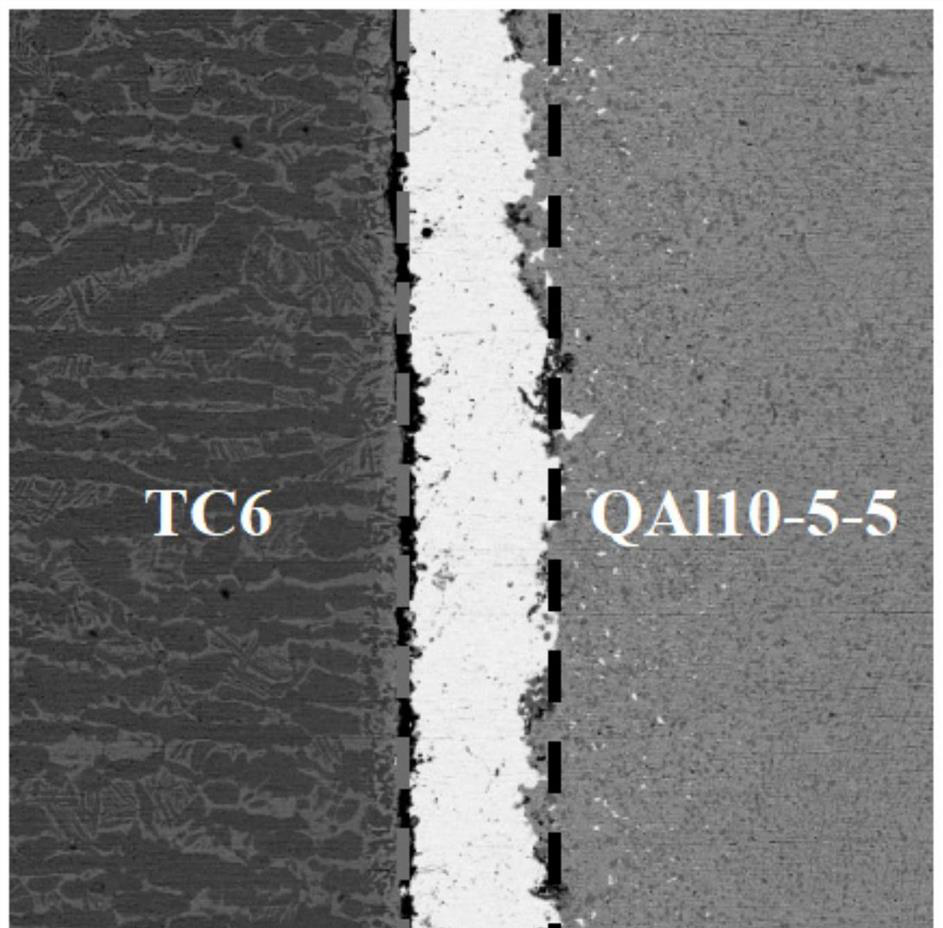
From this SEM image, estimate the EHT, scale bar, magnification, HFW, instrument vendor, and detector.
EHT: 10 kV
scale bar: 30000 nm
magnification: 2 K X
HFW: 134 µm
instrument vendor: Thermo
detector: BSD Full